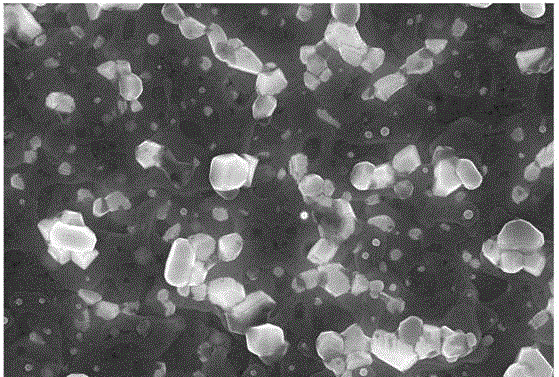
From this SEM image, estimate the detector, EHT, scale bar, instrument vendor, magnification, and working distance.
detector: InLens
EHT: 15 kV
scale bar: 1000 nm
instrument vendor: Zeiss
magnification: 10 K X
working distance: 8.1 mm